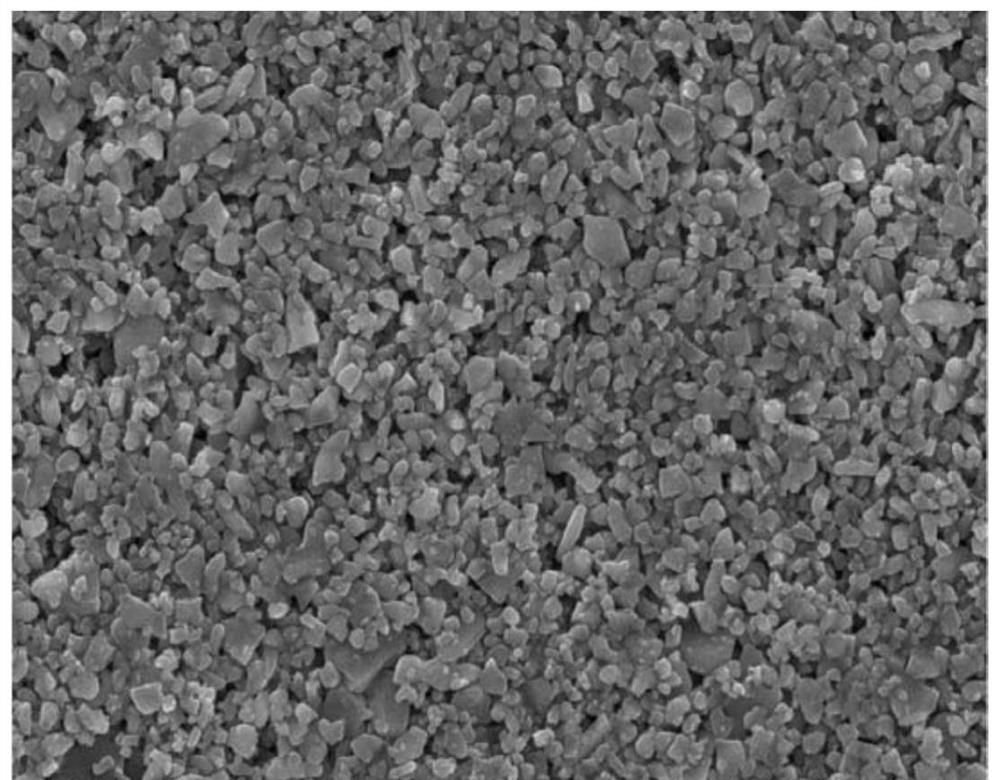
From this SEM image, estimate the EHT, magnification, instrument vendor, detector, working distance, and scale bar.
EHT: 15 kV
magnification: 5 K X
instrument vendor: JEOL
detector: SEI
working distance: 11 mm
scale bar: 5000 nm